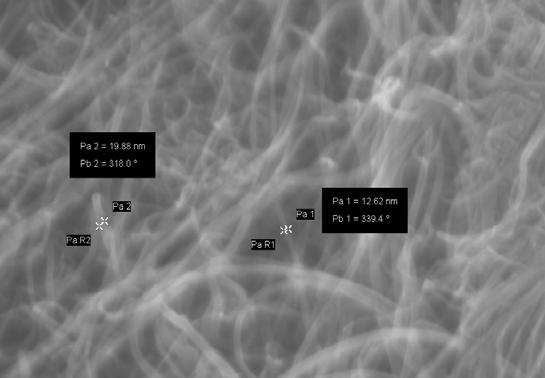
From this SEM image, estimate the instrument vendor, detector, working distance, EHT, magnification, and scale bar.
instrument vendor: Zeiss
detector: InLens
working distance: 3 mm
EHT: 10 kV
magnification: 75.57 K X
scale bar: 200 nm